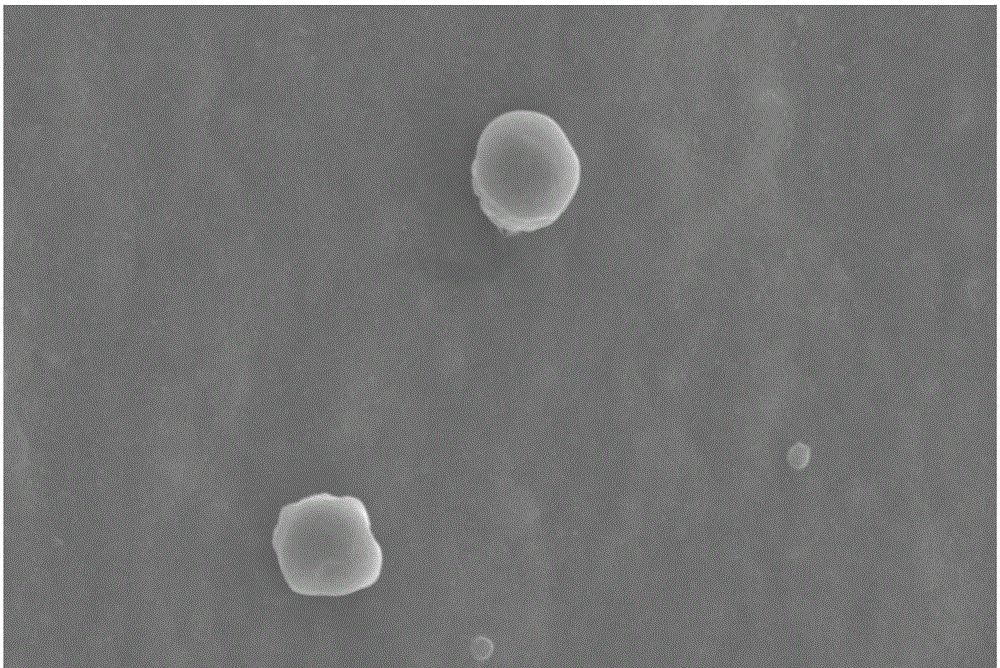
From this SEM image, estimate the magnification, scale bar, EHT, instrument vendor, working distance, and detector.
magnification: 3.5 K X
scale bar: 10000 nm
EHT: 10 kV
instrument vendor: Hitachi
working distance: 13.5 mm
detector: SE